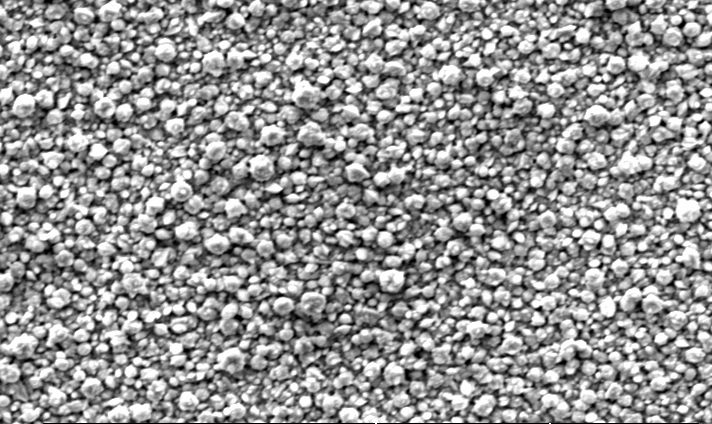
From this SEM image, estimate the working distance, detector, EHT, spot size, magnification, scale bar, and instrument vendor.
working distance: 10.2 mm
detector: SE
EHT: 20 kV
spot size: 6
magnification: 3 K X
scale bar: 20000 nm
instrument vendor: FEI